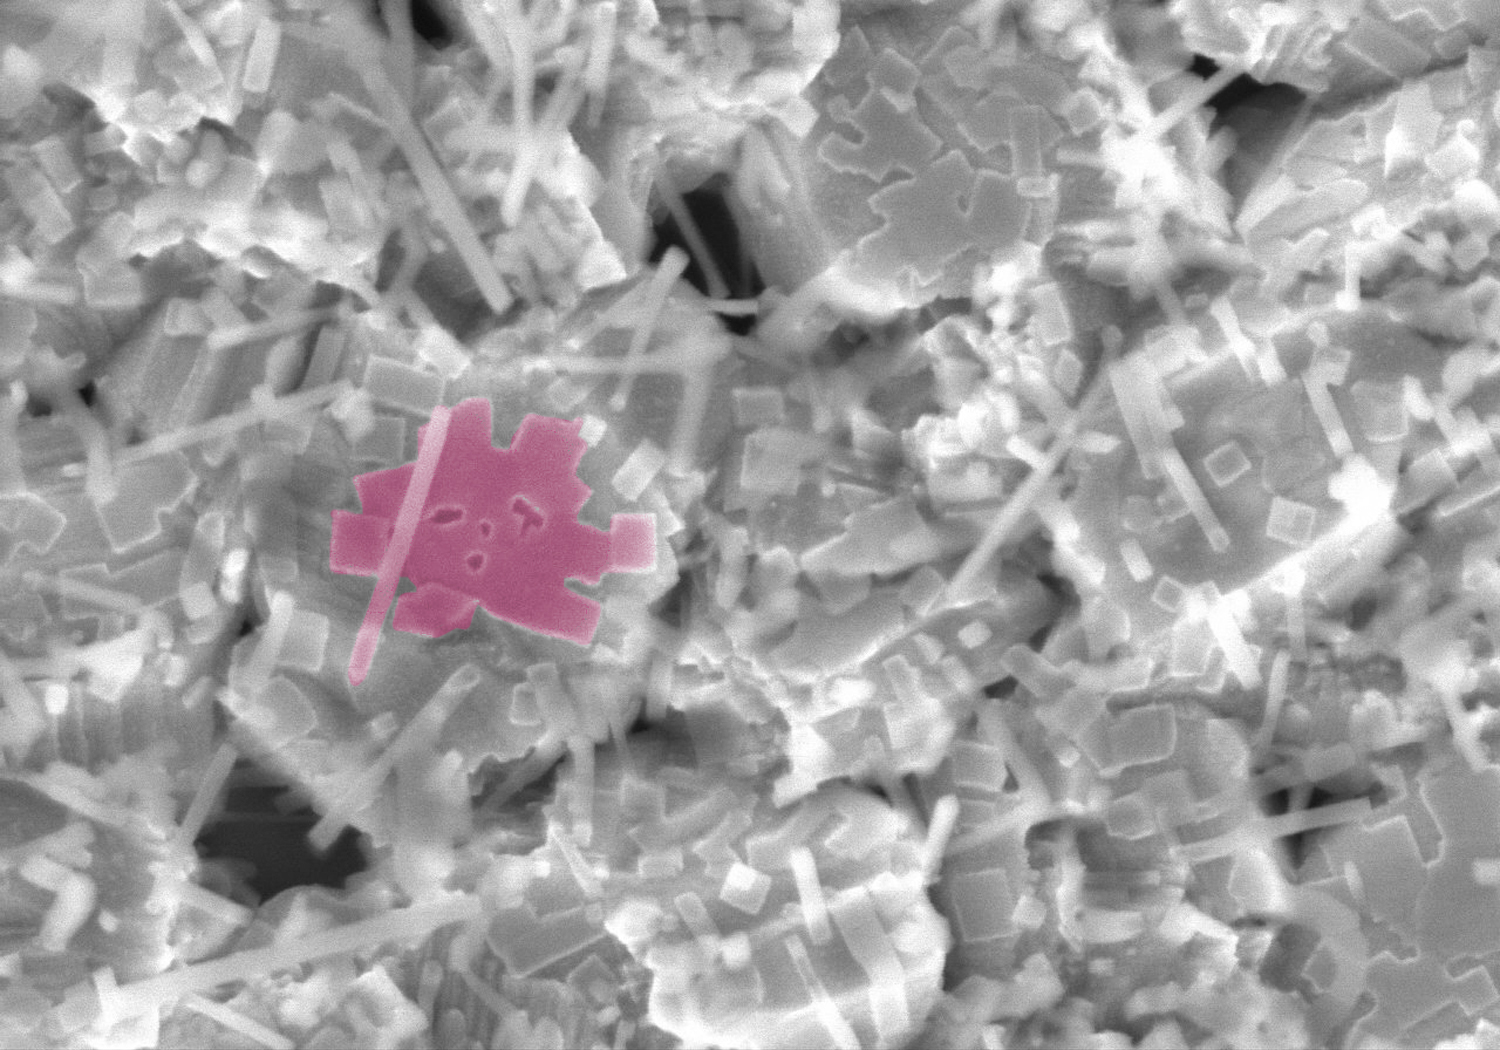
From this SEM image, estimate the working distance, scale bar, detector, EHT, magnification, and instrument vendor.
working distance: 7.7 mm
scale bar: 1000 nm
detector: SE(M)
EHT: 5 kV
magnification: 50 K X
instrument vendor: Hitachi